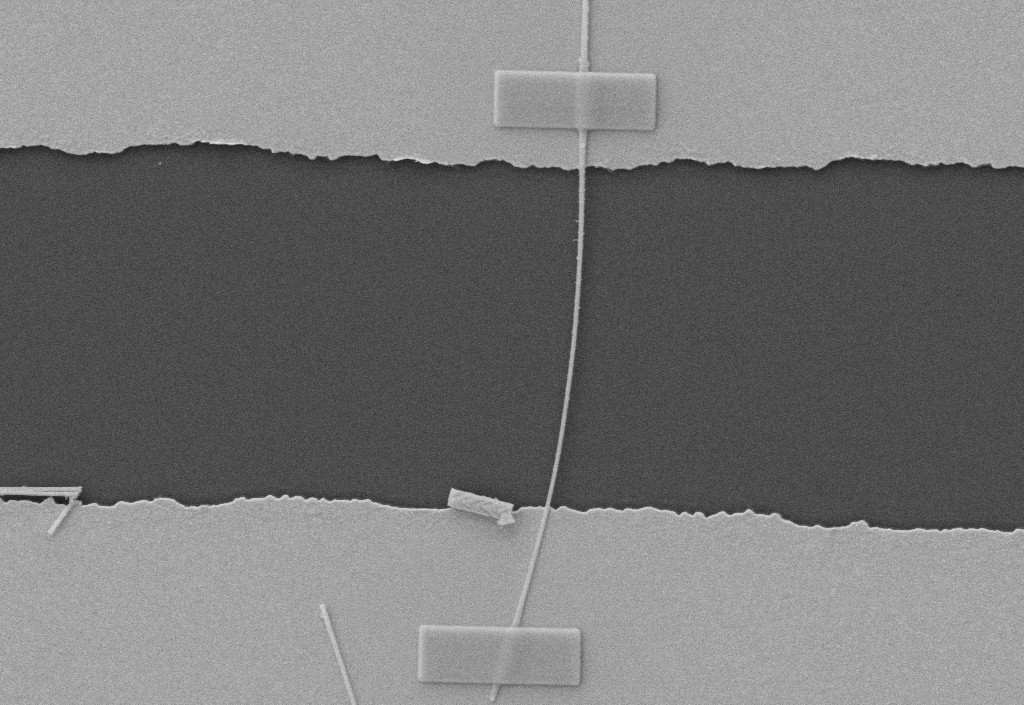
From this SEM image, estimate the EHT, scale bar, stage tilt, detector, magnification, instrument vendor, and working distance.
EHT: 5 kV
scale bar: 1000 nm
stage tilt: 45°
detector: SE2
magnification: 15 K X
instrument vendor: Zeiss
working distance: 5.3 mm